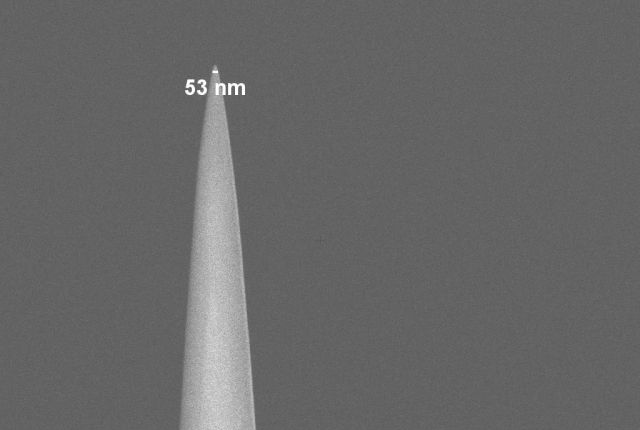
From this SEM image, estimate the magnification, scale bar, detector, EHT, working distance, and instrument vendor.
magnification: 20 K X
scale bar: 2000 nm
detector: InLens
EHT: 2 kV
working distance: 4.9 mm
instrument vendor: Zeiss